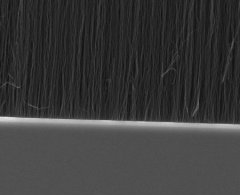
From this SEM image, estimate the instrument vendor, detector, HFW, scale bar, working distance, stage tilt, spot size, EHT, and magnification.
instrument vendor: FEI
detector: ETD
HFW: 19.9 µm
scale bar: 5000 nm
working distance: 13.2 mm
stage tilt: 0°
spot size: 3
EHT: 10 kV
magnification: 15 K X